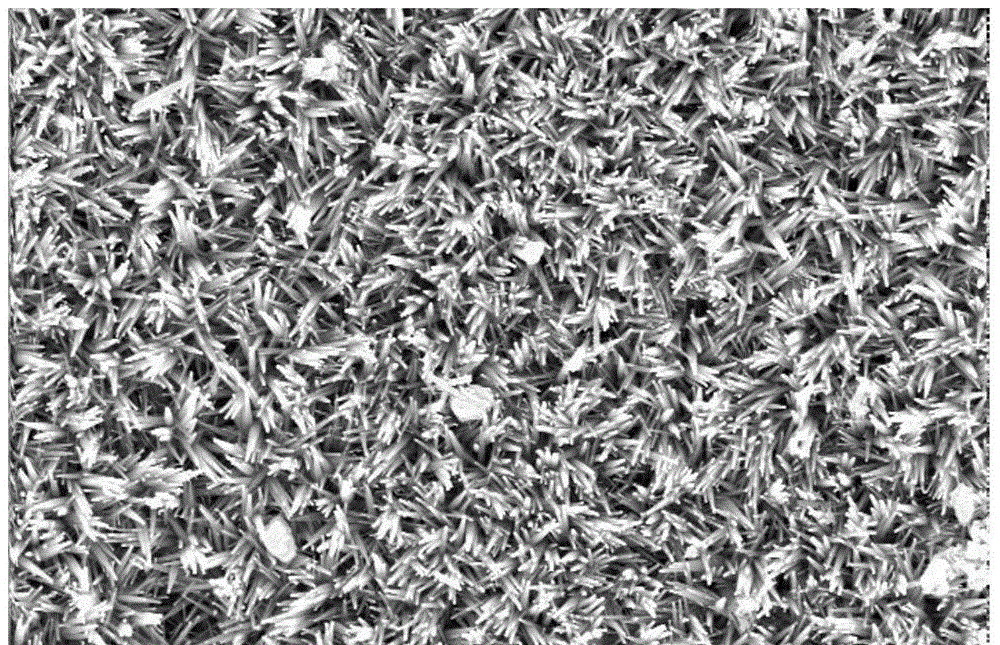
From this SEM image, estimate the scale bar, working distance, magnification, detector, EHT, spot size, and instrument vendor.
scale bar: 5000 nm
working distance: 5.4 mm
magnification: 10 K X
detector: SE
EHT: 20 kV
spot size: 3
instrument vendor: FEI